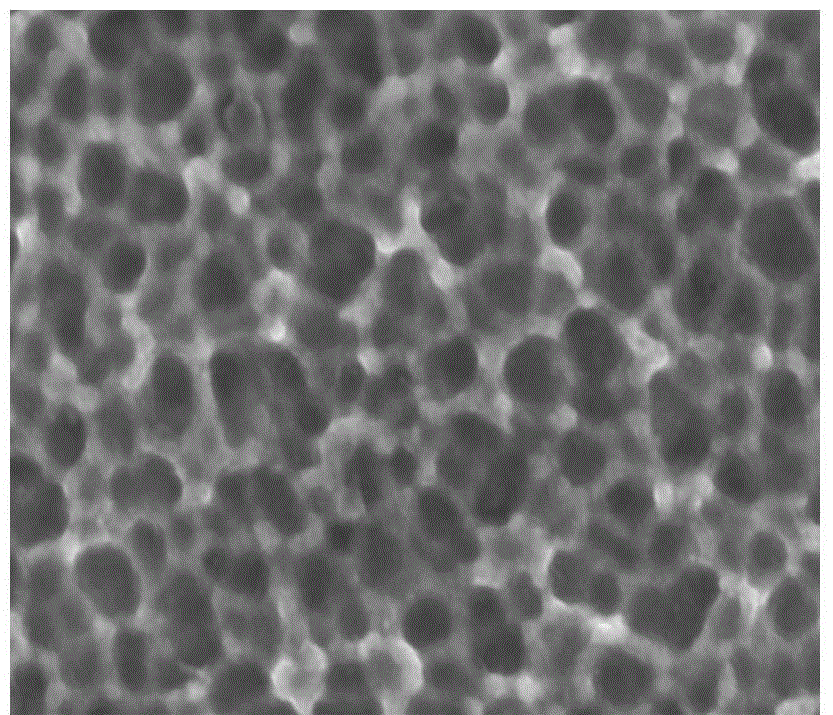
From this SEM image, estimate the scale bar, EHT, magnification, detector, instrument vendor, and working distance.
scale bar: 100 nm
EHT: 5 kV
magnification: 70 K X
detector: SEI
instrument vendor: JEOL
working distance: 6.8 mm